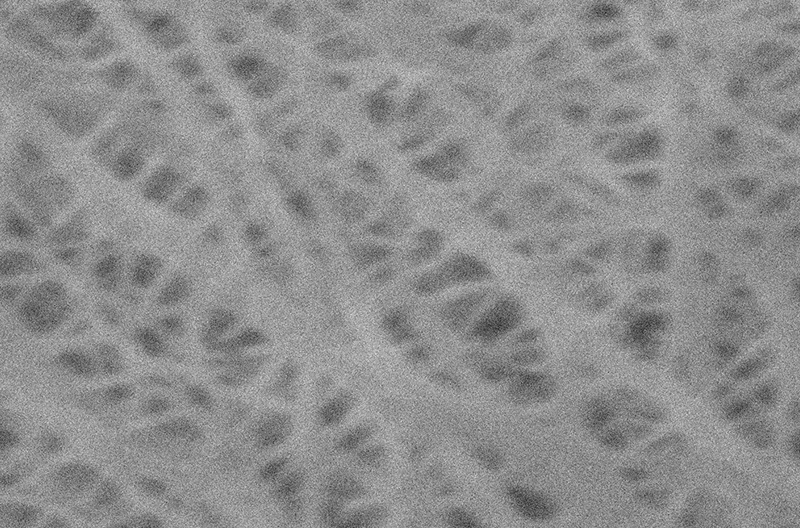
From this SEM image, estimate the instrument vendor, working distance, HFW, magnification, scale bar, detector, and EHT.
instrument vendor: FEI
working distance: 5.77 mm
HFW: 6.41 µm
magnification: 25 K X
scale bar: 500 nm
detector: ETD SE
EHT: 5 kV